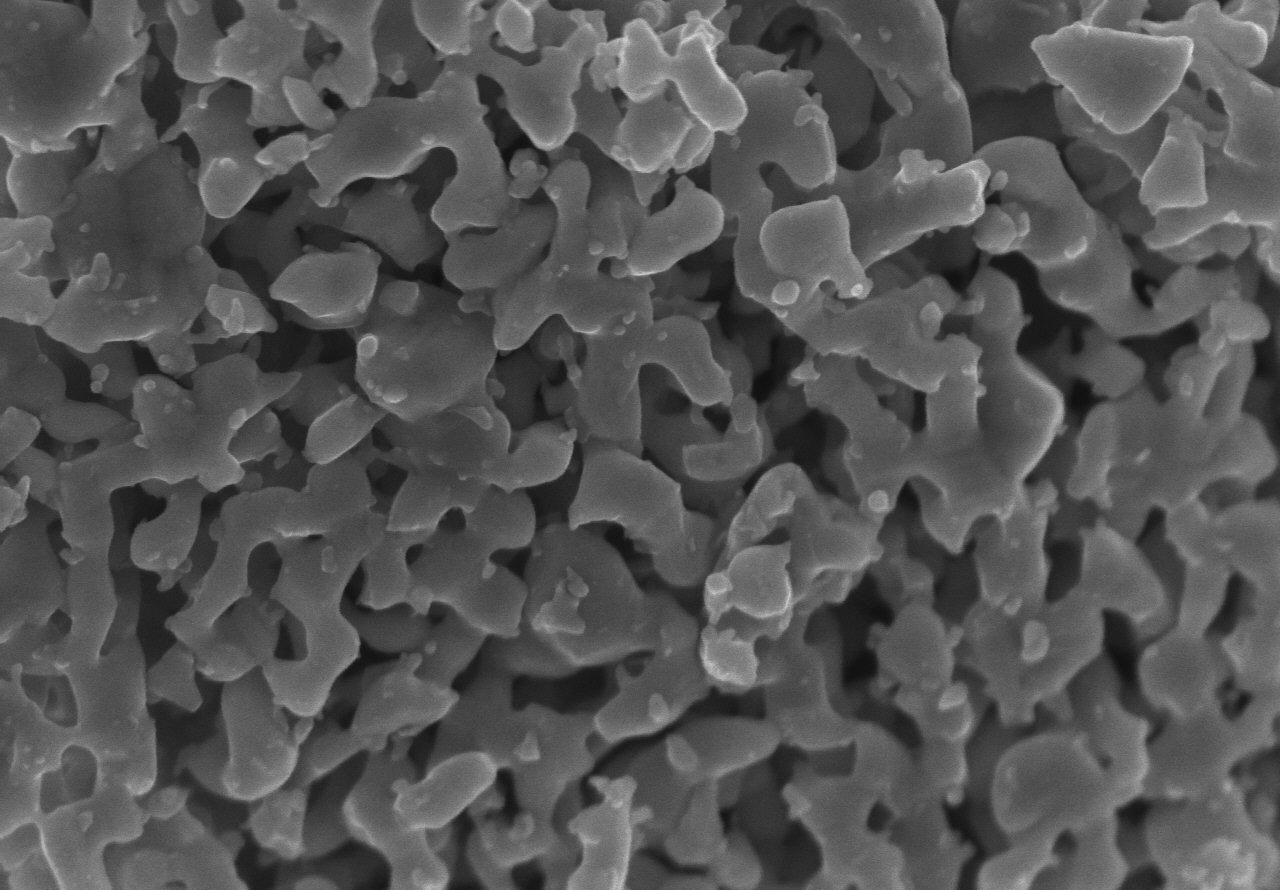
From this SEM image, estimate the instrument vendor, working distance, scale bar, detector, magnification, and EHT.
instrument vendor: Hitachi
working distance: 2.1 mm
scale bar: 1000 nm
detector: SE(U)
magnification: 50 K X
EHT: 5 kV